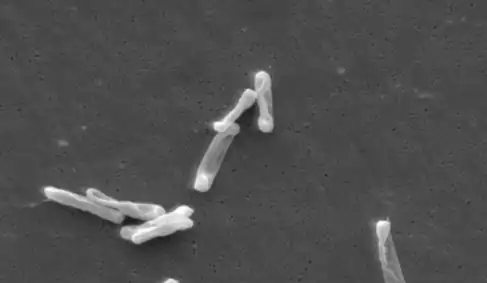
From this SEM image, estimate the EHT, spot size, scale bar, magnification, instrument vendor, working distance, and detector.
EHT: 10 kV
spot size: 3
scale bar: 5000 nm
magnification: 4.753 K X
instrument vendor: FEI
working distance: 33.8 mm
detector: SE